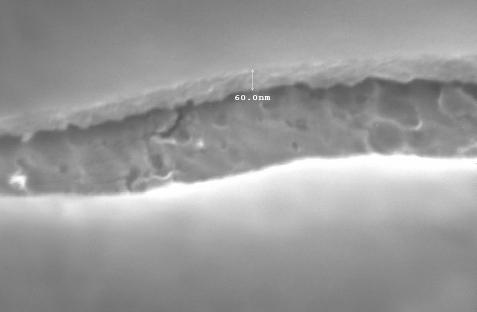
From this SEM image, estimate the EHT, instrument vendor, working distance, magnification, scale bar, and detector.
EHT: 10 kV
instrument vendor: JEOL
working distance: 10.2 mm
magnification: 100 K X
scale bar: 100 nm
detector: SEI